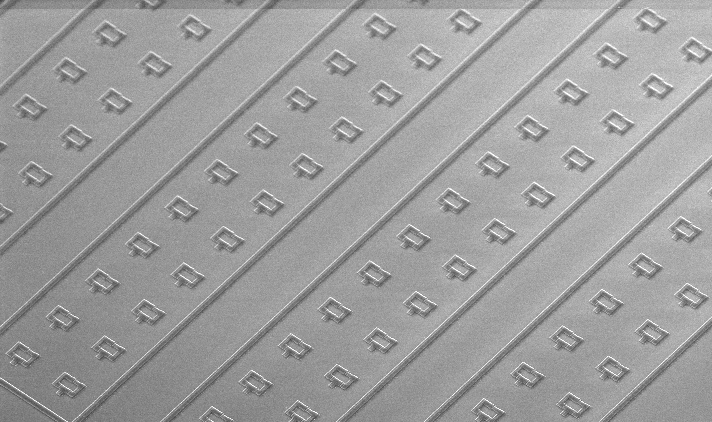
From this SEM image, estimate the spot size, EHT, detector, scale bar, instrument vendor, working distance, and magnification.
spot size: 3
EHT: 5 kV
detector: TLD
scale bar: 10000 nm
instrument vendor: FEI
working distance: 5.4 mm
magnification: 4.535 K X